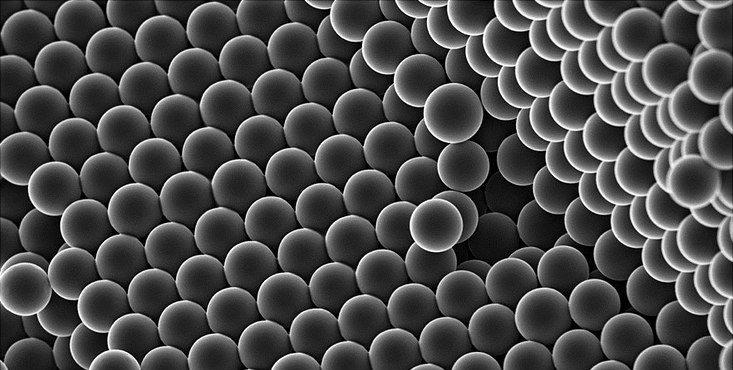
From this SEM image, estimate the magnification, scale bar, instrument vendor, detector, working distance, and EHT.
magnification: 10 K X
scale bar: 1000 nm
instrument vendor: Zeiss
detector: InLens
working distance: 4.8 mm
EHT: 5 kV